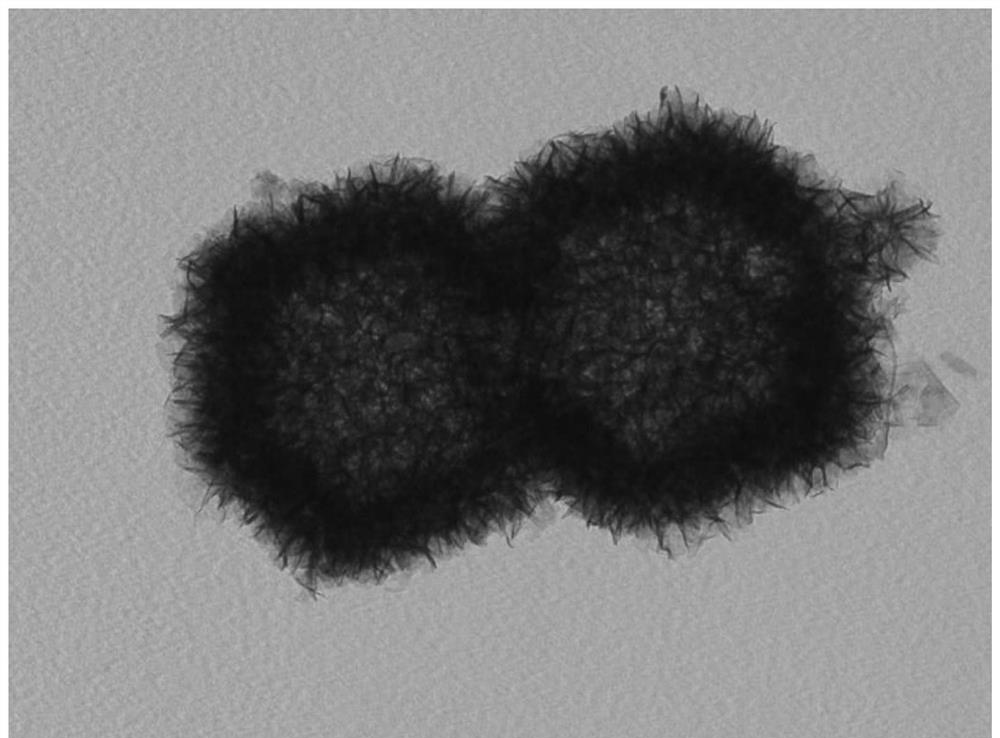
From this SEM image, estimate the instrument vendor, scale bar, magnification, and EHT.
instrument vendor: JEOL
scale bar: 500 nm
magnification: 60 K X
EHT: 100 kV